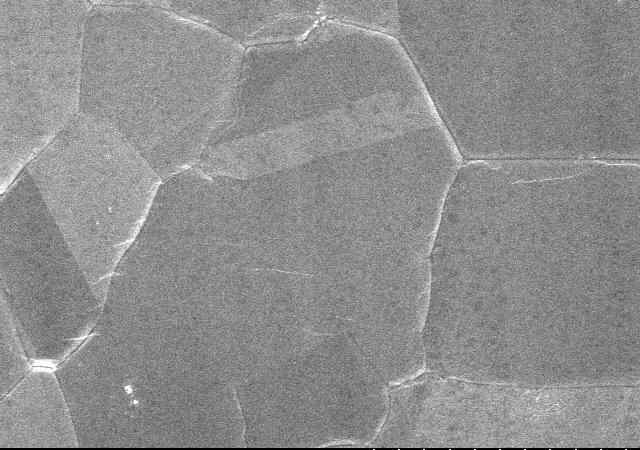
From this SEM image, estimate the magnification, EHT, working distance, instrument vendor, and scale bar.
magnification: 1 K X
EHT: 15 kV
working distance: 9.7 mm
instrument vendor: Hitachi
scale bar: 50000 nm